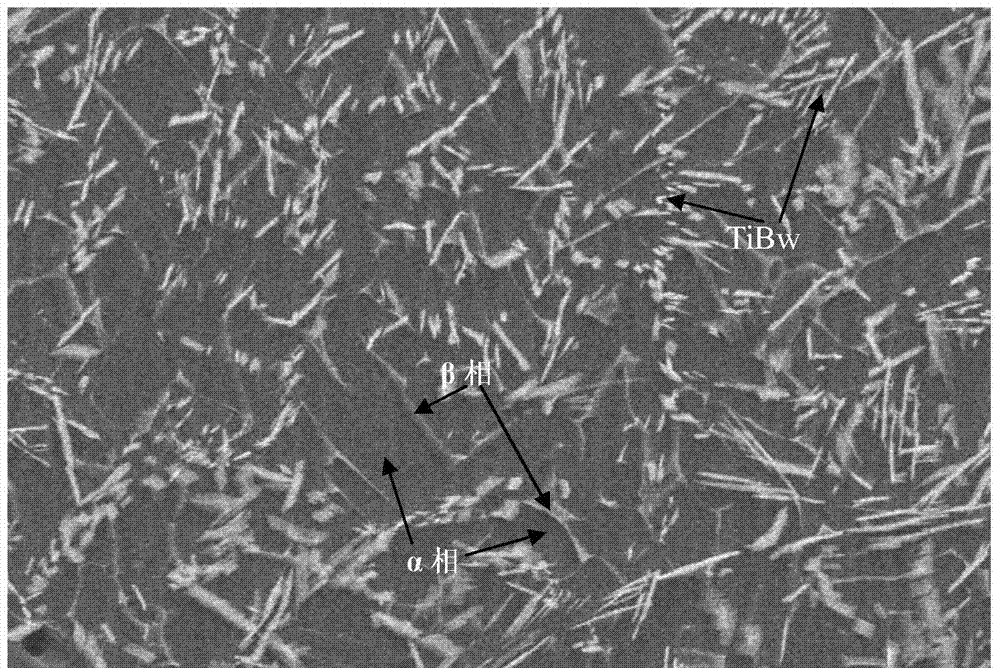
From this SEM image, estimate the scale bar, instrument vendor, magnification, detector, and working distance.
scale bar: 50000 nm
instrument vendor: Hitachi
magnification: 1 K X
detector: SE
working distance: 22.3 mm